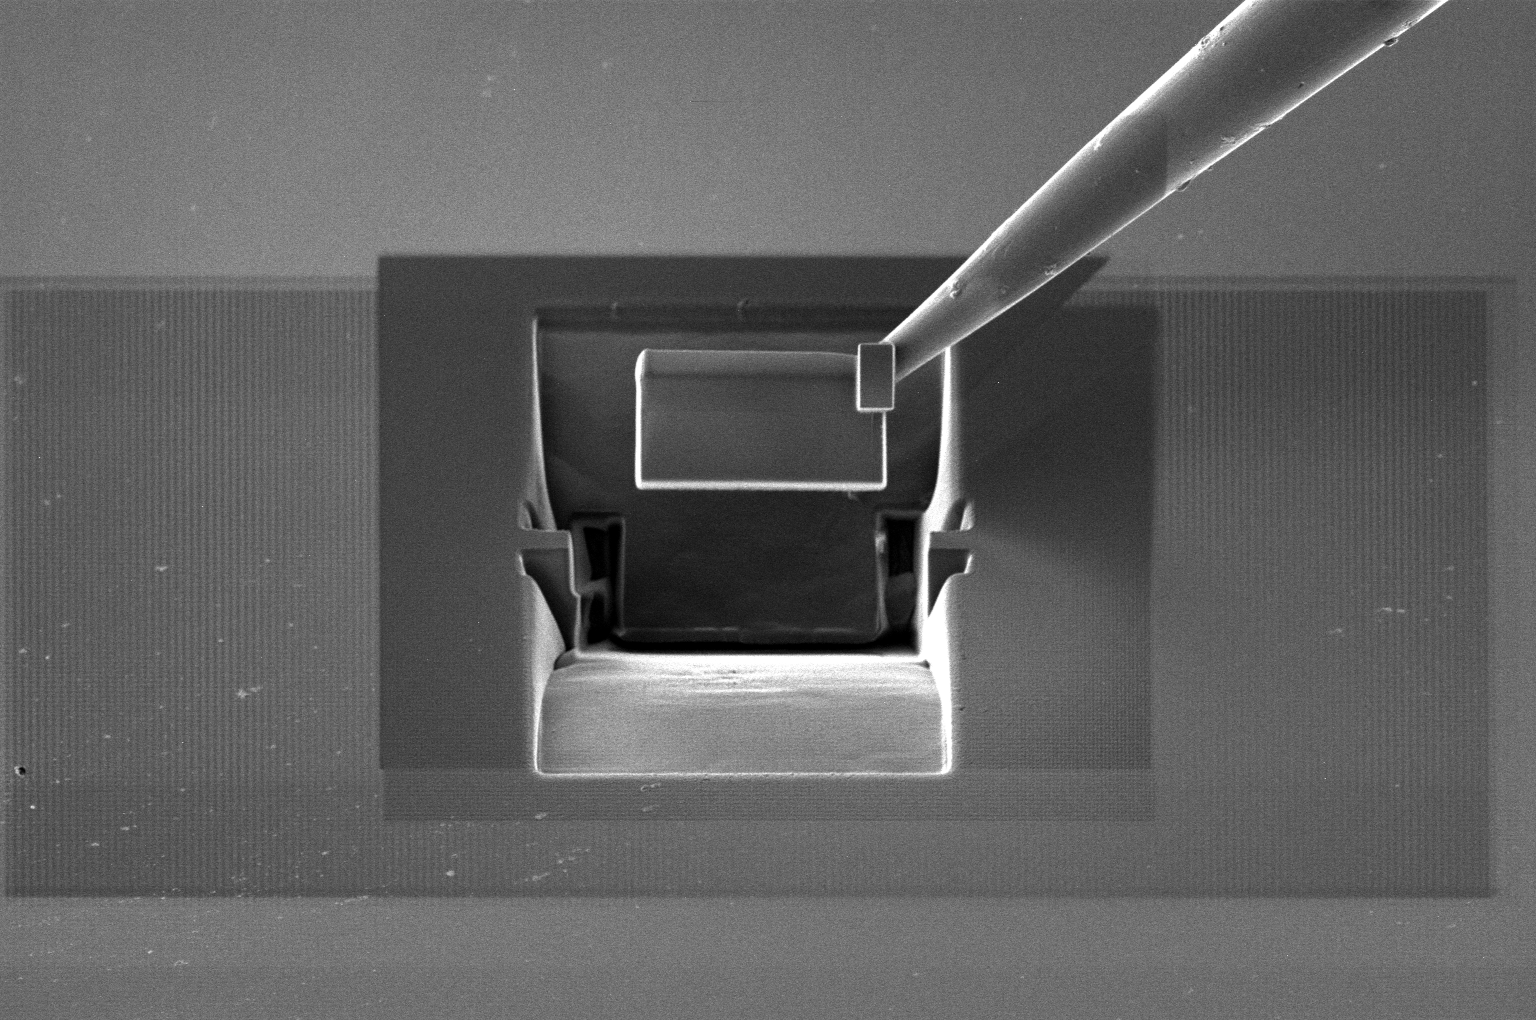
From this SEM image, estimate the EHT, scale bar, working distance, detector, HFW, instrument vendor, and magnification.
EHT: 30 kV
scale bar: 20000 nm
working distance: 11 mm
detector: ETD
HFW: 82.9 µm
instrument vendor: Thermo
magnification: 5 K X